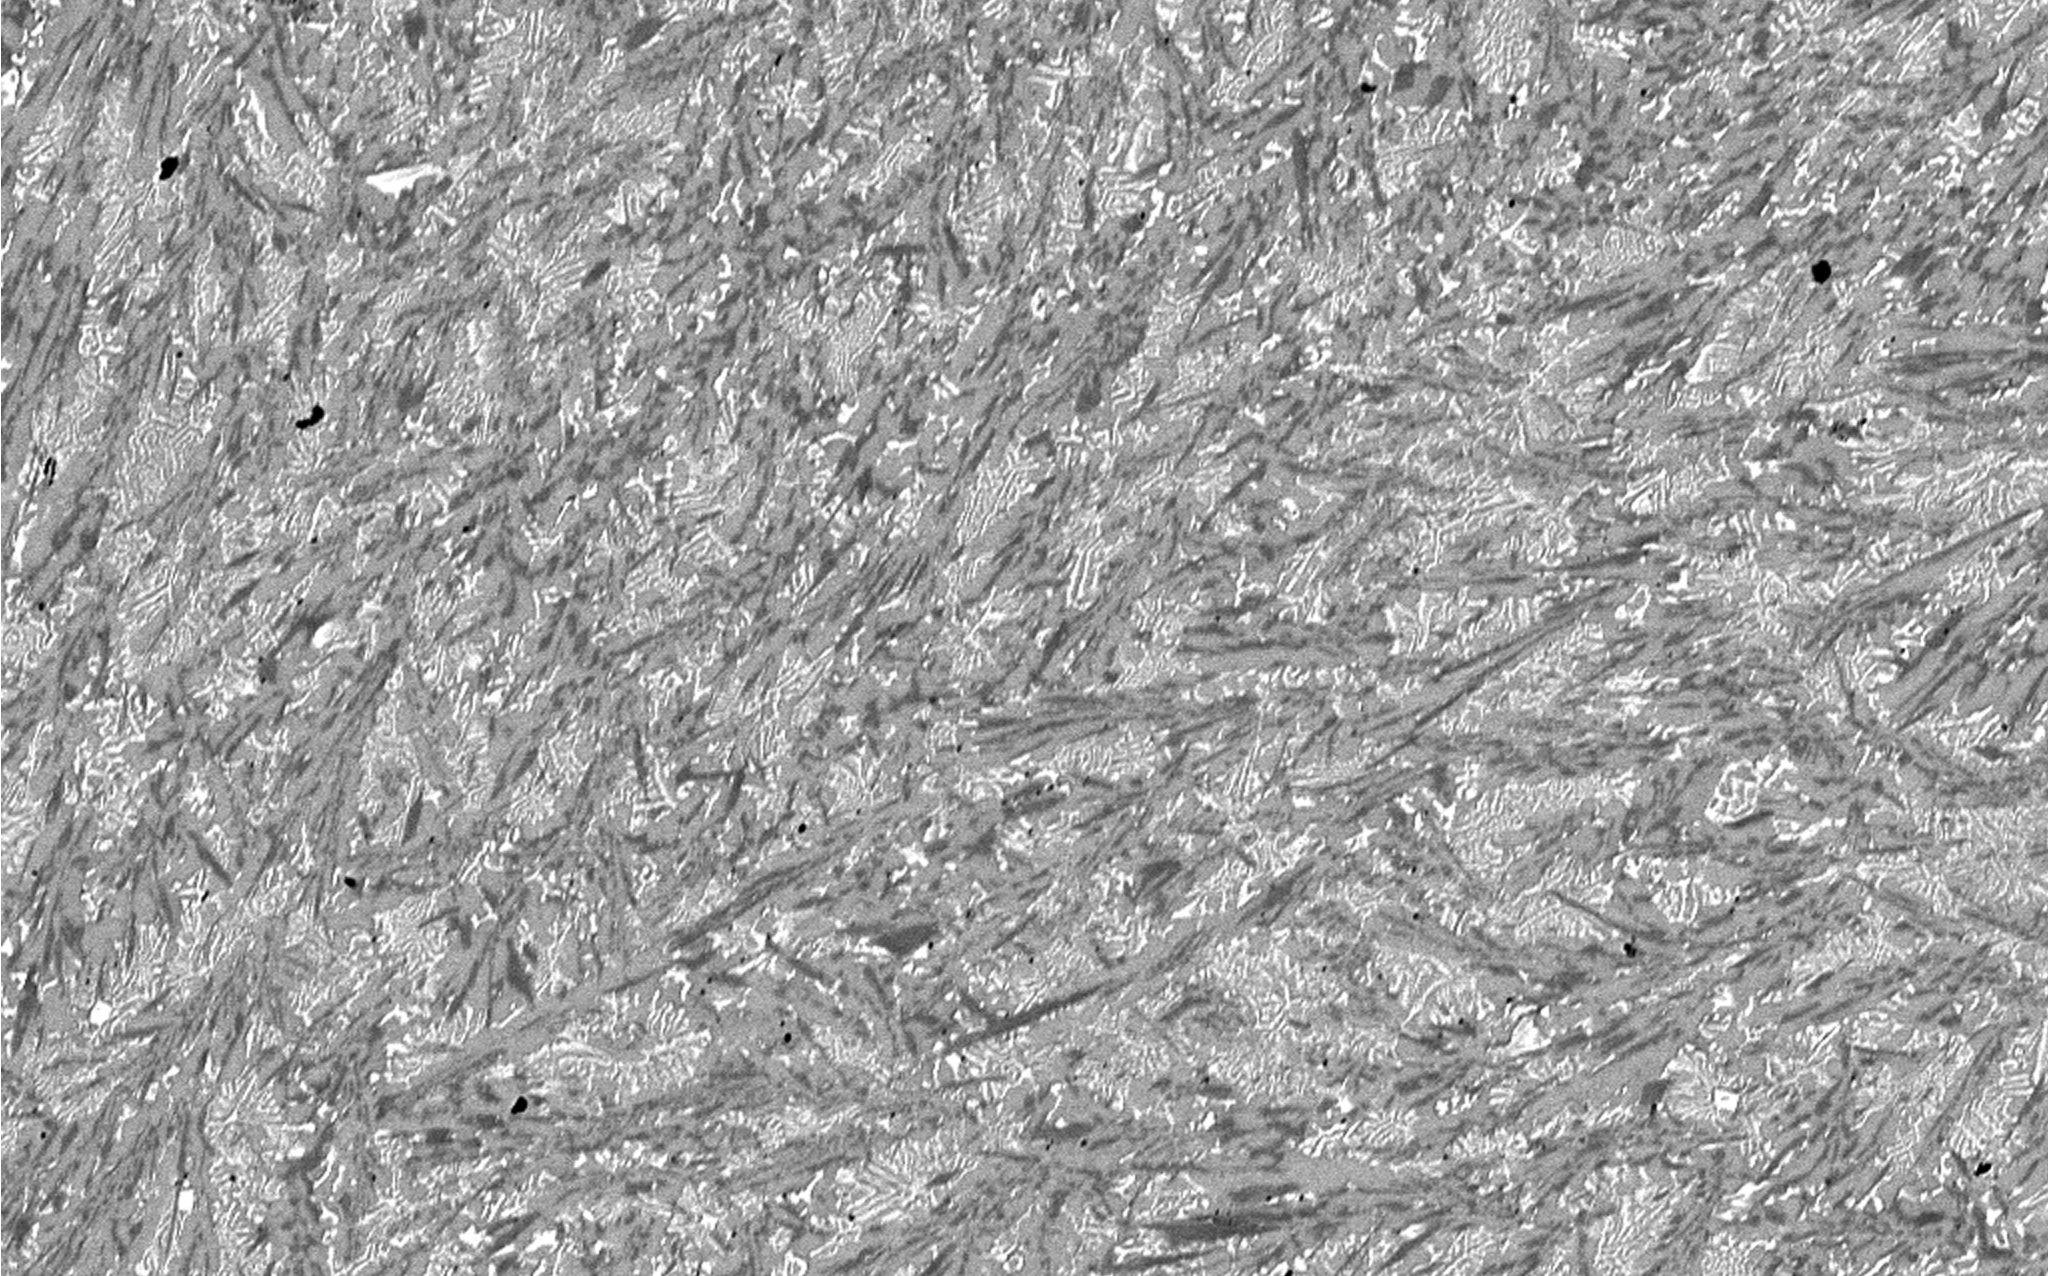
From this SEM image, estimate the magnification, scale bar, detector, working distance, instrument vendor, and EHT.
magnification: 1 K X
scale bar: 50000 nm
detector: BSE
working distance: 10.77 mm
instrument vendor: Tescan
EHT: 20 kV